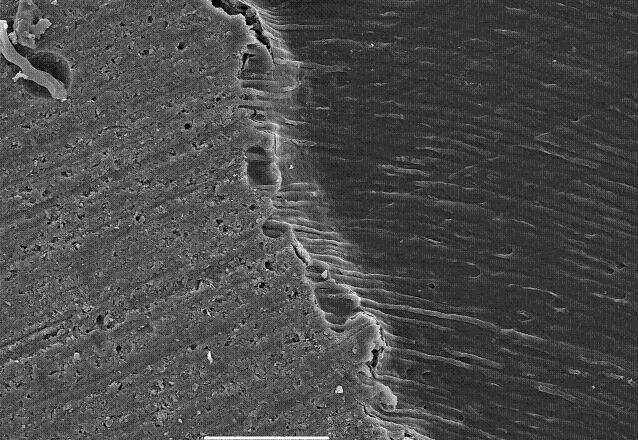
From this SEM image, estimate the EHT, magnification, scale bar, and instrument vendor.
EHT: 15 kV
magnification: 0.5 K X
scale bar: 50000 nm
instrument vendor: JEOL